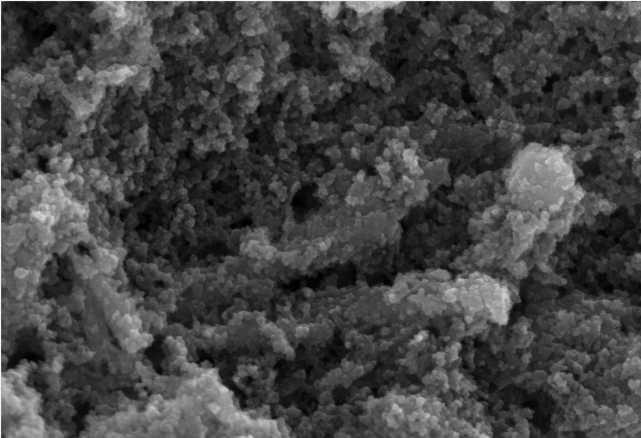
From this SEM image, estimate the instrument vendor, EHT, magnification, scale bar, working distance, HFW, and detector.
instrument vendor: Zeiss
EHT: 5 kV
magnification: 86.63 K X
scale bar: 200 nm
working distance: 7.2 mm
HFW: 3.244 µm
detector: InLens